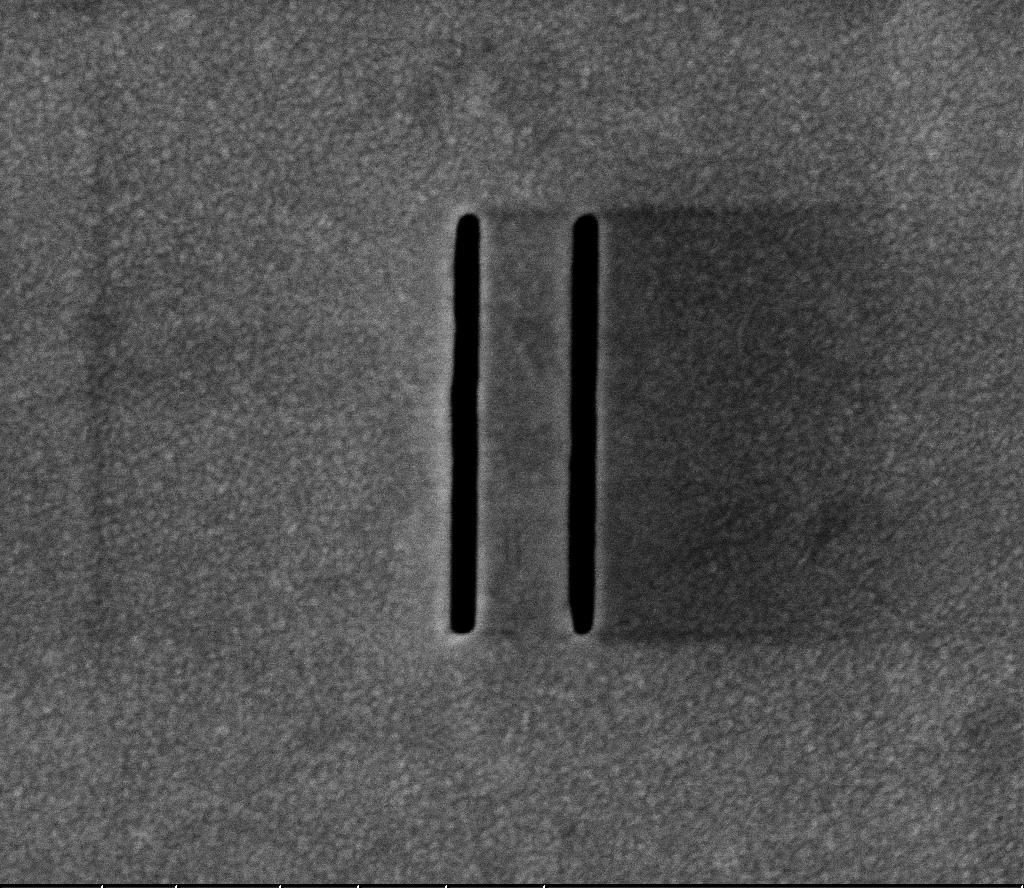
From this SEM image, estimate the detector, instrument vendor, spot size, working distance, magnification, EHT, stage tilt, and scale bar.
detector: TLD-S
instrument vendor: FEI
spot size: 3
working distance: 5.004 mm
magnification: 80 K X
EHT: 5 kV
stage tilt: -0°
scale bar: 1000 nm